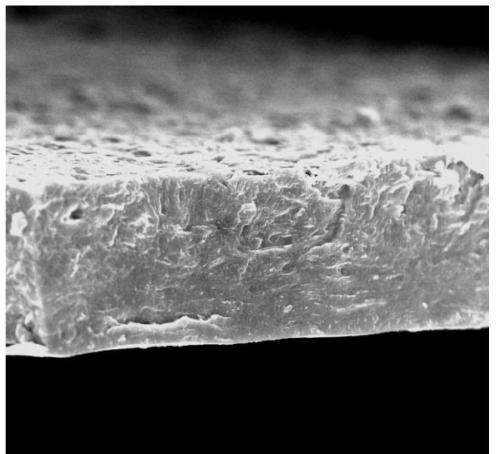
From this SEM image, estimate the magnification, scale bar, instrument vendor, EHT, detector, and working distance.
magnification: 5 K X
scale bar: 20000 nm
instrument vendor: FEI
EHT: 10 kV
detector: TLD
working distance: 5.5 mm